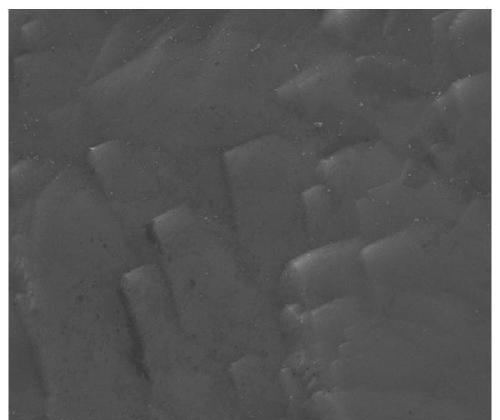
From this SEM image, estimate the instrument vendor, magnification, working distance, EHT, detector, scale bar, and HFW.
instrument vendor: FEI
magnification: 5 K X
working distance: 9.1 mm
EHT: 20 kV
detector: ETD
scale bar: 20000 nm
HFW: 59.7 µm